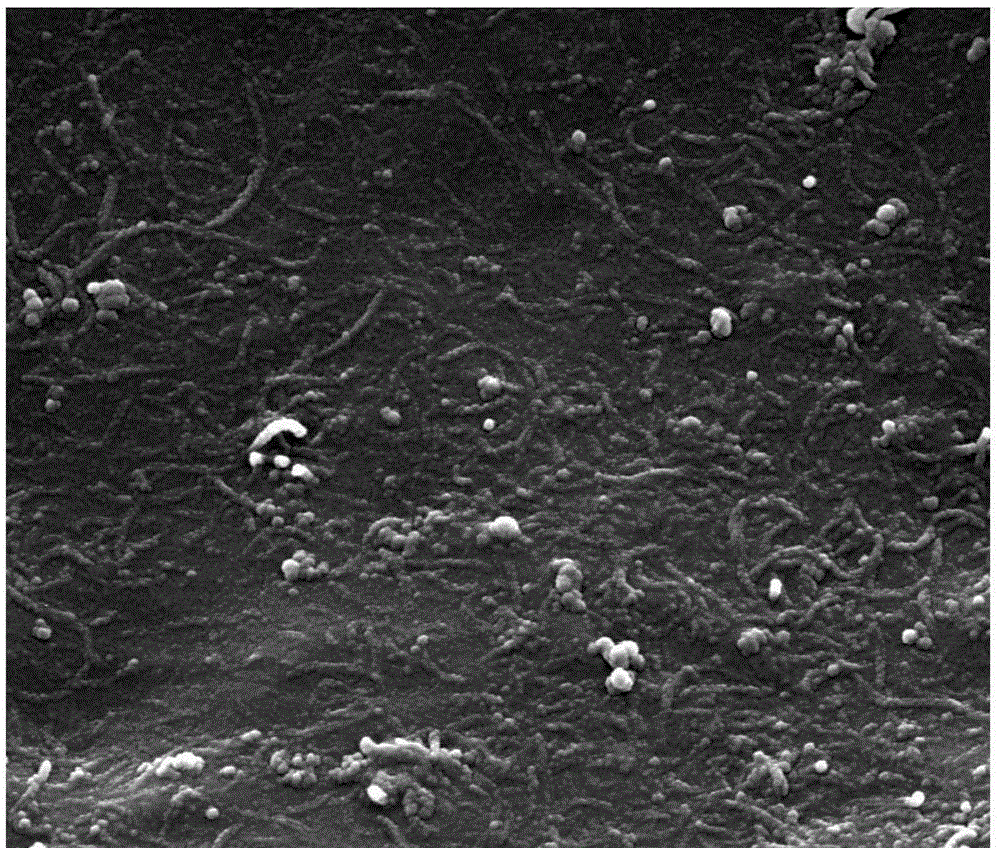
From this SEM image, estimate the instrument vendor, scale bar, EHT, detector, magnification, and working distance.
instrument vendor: FEI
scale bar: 1000 nm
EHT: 20 kV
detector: SE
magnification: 80 K X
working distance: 13 mm